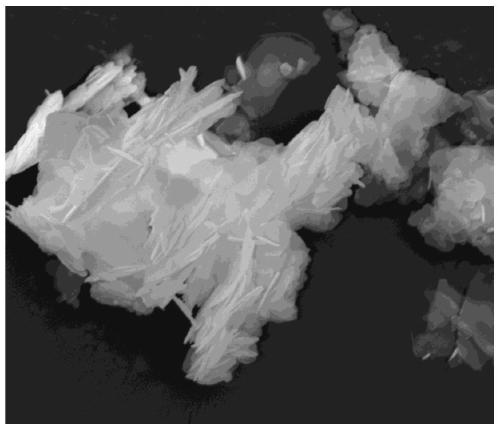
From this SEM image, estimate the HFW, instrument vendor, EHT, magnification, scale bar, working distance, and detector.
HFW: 14.9 µm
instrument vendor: FEI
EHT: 20 kV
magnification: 20 K X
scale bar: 5000 nm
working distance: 12 mm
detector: ETD